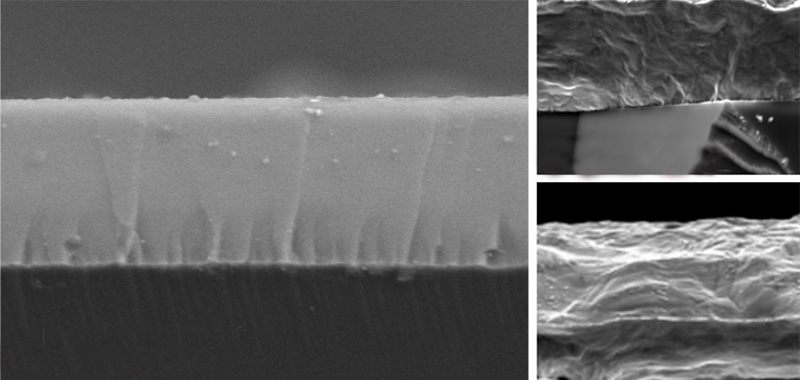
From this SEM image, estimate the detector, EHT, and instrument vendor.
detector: SE2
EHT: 15 kV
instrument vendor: Zeiss